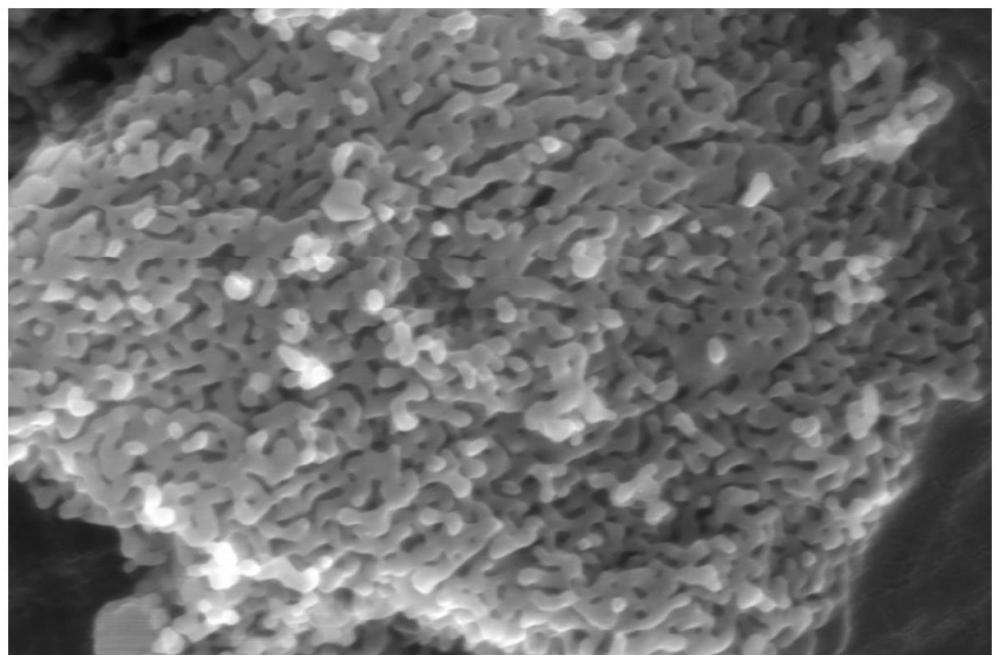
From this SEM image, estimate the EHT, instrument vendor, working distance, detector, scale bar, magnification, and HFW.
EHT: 20 kV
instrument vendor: Thermo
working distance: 11.1575 mm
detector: ETD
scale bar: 500 nm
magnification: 100 K X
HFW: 4.14 µm